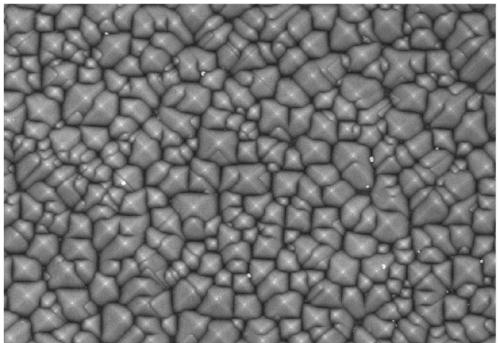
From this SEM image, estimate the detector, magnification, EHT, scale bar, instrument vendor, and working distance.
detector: SE(M)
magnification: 10 K X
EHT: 5 kV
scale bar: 5000 nm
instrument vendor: Hitachi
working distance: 5.1 mm